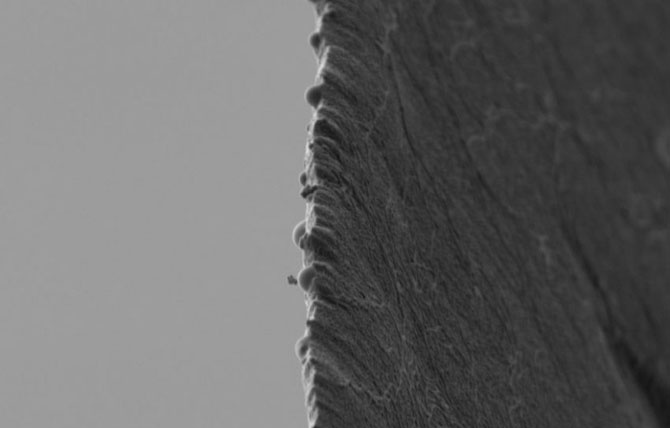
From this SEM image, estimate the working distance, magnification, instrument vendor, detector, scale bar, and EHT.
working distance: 6.1 mm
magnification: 10 K X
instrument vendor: Zeiss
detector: SE2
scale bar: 1000 nm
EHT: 1 kV